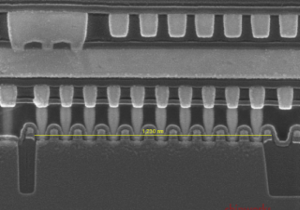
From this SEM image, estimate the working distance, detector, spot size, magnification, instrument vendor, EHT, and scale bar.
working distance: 5.1 mm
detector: TLD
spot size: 3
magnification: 80 K X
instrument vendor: FEI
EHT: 10 kV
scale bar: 200 nm